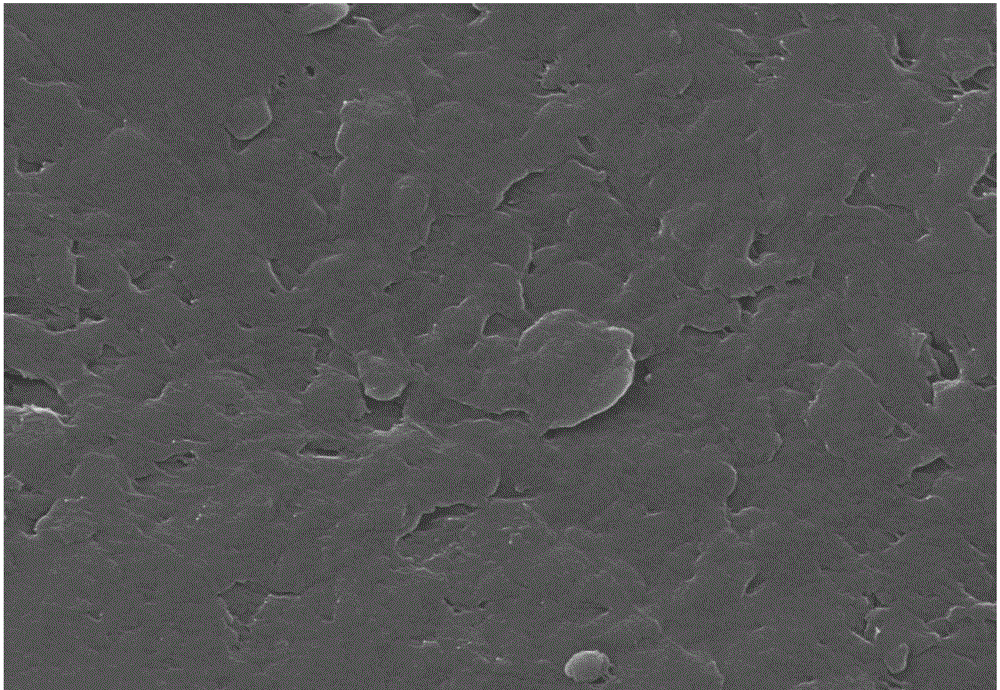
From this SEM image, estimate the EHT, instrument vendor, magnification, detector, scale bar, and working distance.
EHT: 20 kV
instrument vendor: Zeiss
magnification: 100 K X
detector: InLens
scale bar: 200 nm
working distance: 5.8 mm